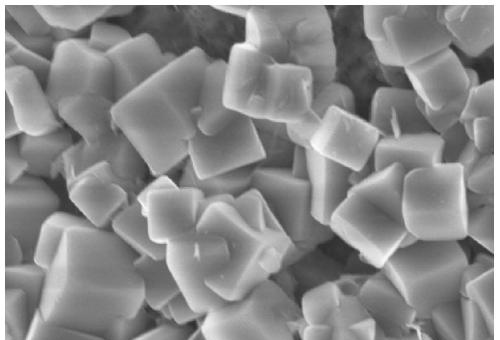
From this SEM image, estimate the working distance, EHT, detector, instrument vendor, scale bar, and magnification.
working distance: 8.6 mm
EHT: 5 kV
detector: SE(UL)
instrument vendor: Hitachi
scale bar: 2000 nm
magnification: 20 K X